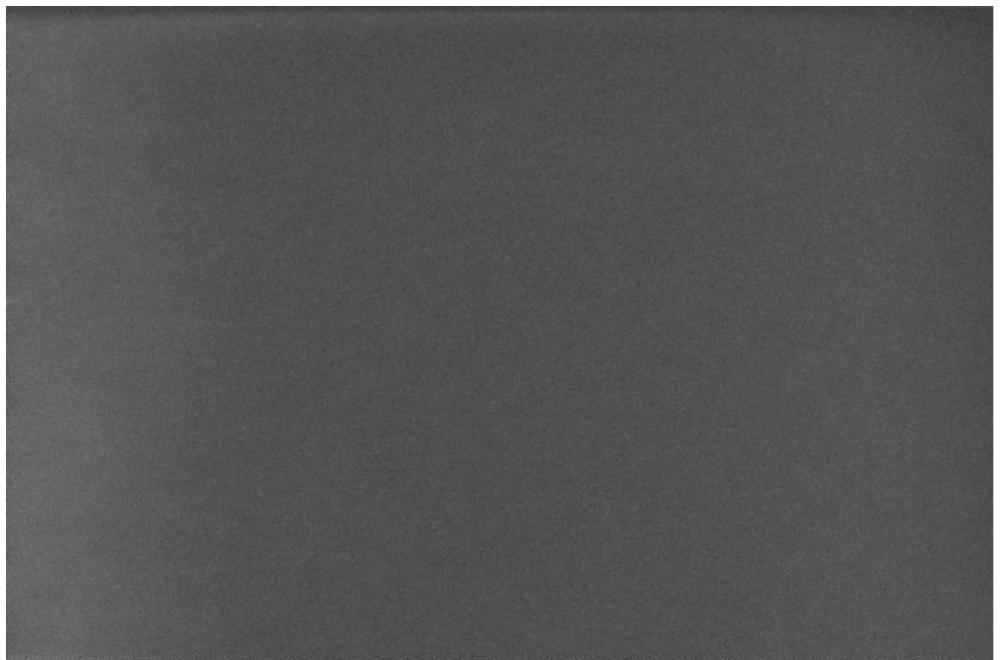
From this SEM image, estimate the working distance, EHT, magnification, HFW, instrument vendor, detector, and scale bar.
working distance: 5.4 mm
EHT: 5 kV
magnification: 5 K X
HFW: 25.4 µm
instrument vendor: Thermo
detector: TLD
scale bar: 5000 nm